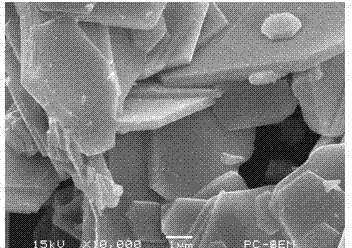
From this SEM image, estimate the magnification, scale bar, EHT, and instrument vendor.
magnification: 10 K X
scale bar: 1000 nm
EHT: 15 kV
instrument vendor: JEOL